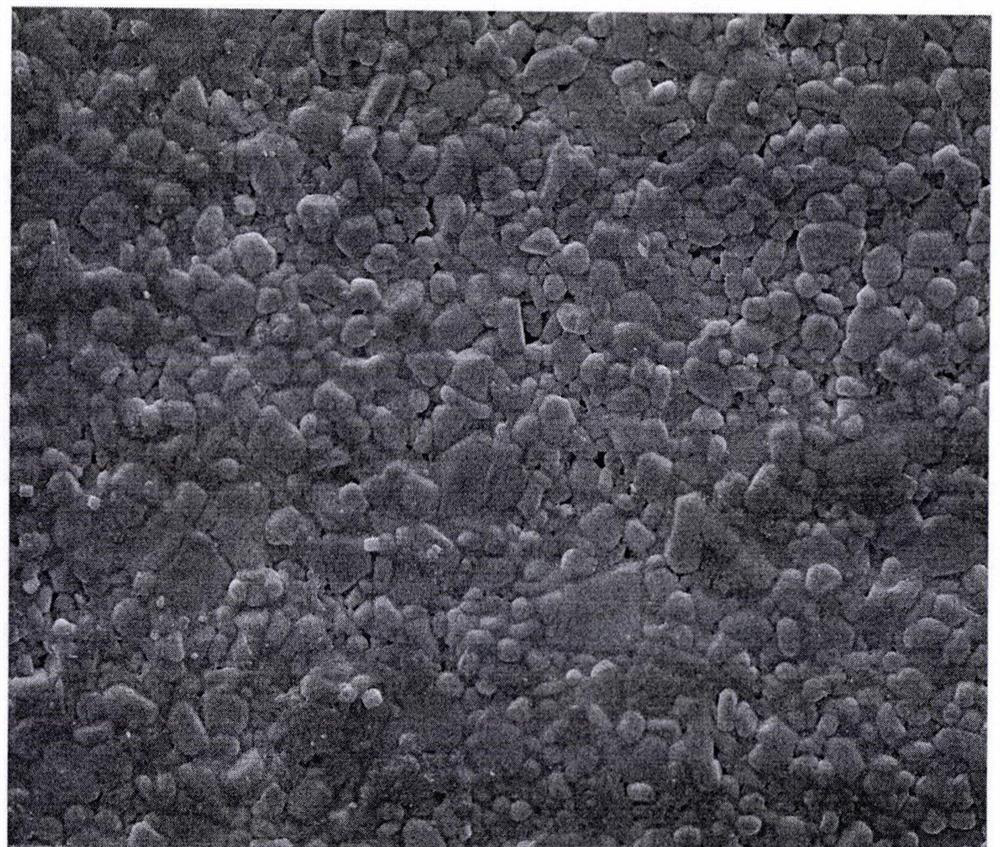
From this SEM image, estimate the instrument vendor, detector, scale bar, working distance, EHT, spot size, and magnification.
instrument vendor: FEI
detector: ETD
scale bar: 10000 nm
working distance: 4.1 mm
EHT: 10 kV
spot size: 3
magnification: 5 K X